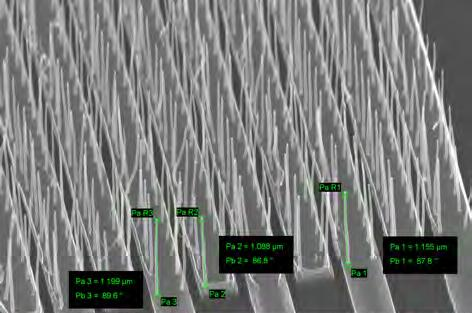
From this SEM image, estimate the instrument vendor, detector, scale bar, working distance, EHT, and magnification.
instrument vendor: Zeiss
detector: InLens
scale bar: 1000 nm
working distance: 3.2 mm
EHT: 15 kV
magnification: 46.67 K X